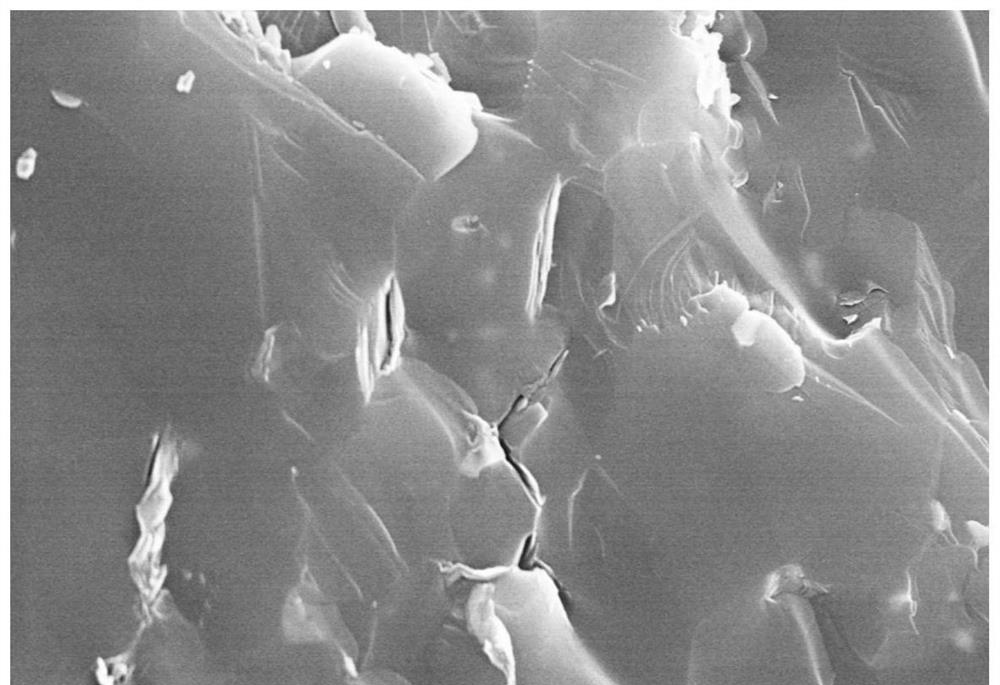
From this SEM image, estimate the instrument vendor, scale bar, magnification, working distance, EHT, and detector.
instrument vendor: Hitachi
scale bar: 5000 nm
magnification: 10 K X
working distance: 7 mm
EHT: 15 kV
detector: SE(UL)